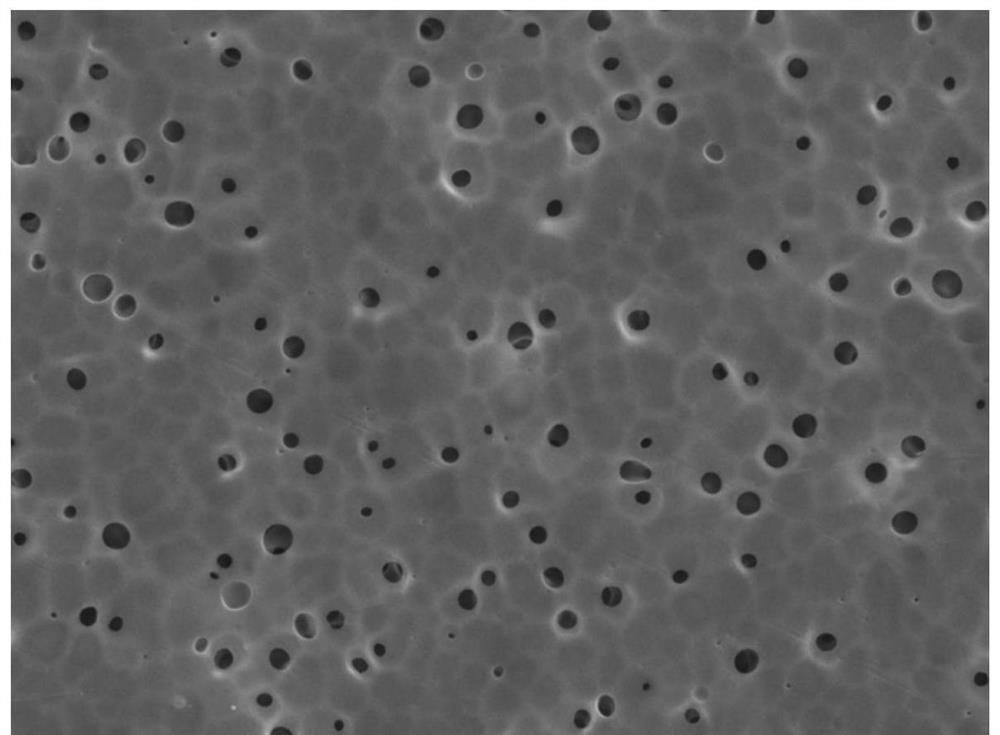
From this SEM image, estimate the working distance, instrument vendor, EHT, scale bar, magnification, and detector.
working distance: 10.3 mm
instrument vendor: JEOL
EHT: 10 kV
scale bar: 10000 nm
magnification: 2 K X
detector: LED